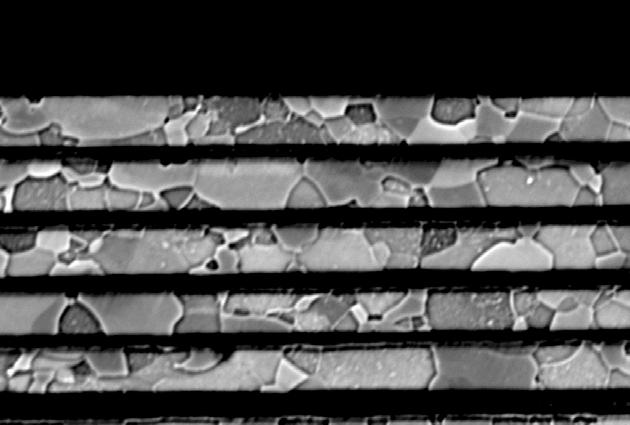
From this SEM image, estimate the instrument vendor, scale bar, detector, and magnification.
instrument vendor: Zeiss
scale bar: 2000 nm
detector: QBSD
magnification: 4 K X